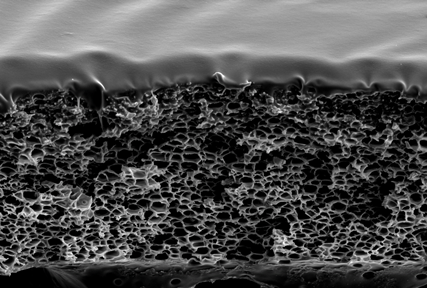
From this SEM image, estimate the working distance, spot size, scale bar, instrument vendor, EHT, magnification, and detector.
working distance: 11 mm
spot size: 50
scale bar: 10000 nm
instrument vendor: JEOL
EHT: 15 kV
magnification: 1.8 K X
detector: SEI